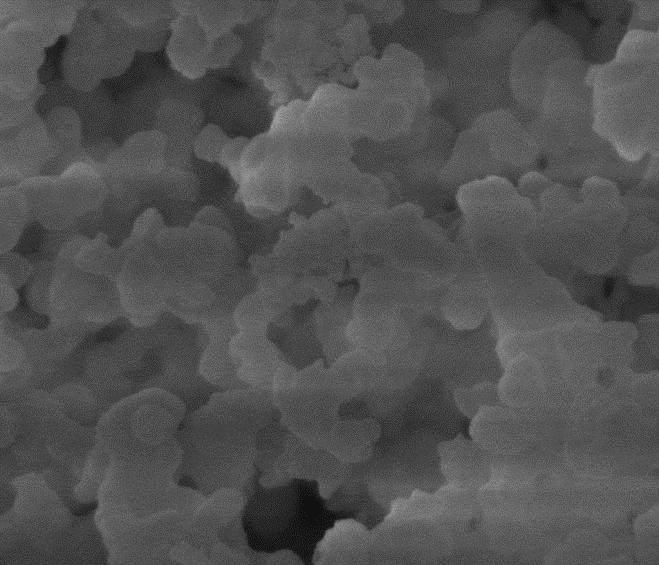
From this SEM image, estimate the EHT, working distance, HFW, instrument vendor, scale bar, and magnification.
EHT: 10 kV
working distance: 5.1 mm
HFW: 3.73 µm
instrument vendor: FEI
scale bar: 1000 nm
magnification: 80 K X